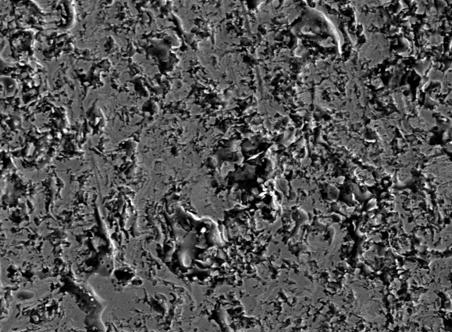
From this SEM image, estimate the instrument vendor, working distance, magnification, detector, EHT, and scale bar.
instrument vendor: Tescan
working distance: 18.383 mm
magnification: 1 K X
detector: SE Detector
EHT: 30 kV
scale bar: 50000 nm